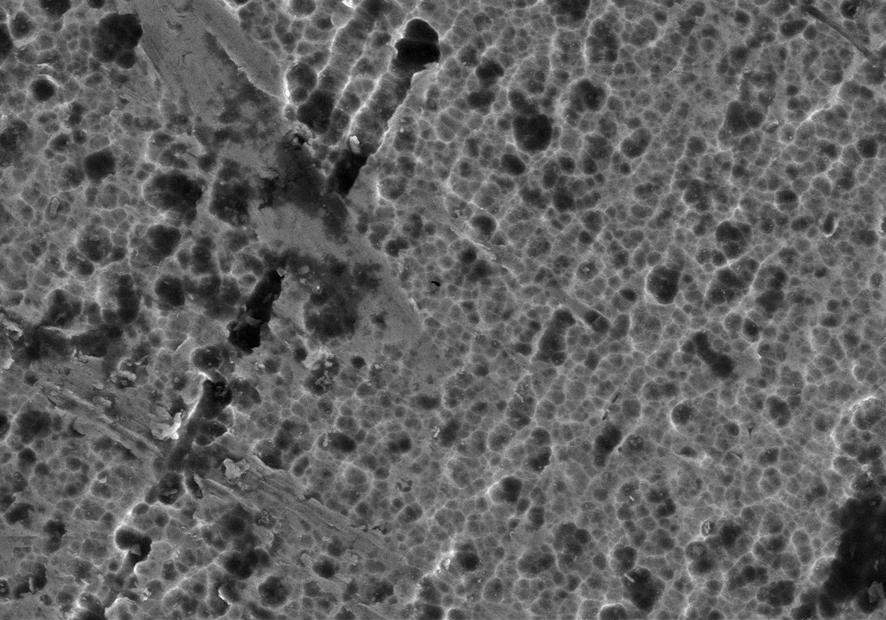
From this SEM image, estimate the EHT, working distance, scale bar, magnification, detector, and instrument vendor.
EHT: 10 kV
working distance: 12.6 mm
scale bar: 10000 nm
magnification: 4.51 K X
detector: SE(M)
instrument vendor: Hitachi